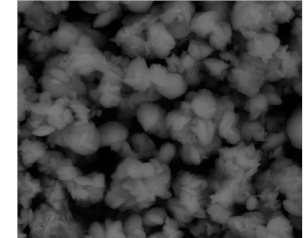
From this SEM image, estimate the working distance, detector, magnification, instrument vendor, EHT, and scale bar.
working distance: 14.9 mm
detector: GAD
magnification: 10 K X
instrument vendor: FEI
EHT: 20 kV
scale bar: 5000 nm